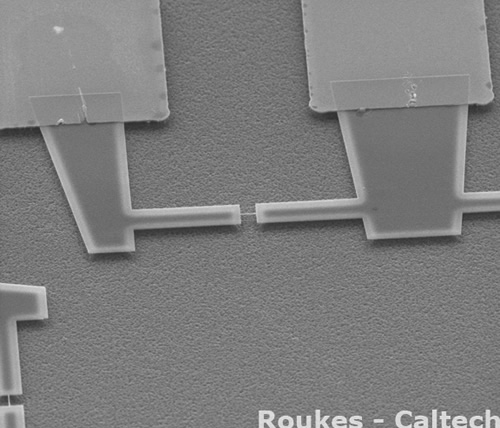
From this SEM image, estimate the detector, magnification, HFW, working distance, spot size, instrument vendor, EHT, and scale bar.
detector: ETD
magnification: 2.5 K X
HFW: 54.08 µm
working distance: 6.8 mm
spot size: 3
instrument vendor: FEI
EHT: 10 kV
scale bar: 20000 nm